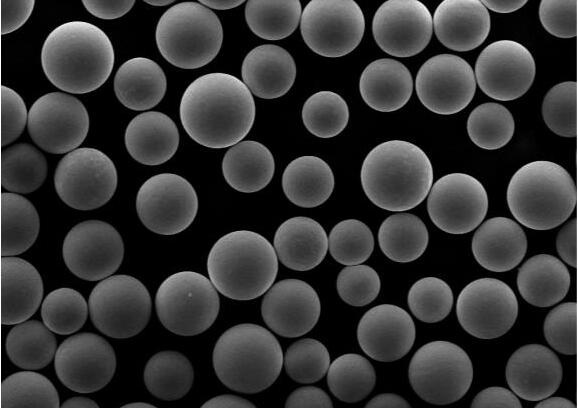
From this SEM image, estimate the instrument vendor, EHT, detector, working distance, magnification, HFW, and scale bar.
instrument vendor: FEI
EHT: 20 kV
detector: ETD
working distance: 8.9 mm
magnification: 0.4 K X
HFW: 317 µm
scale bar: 100000 nm